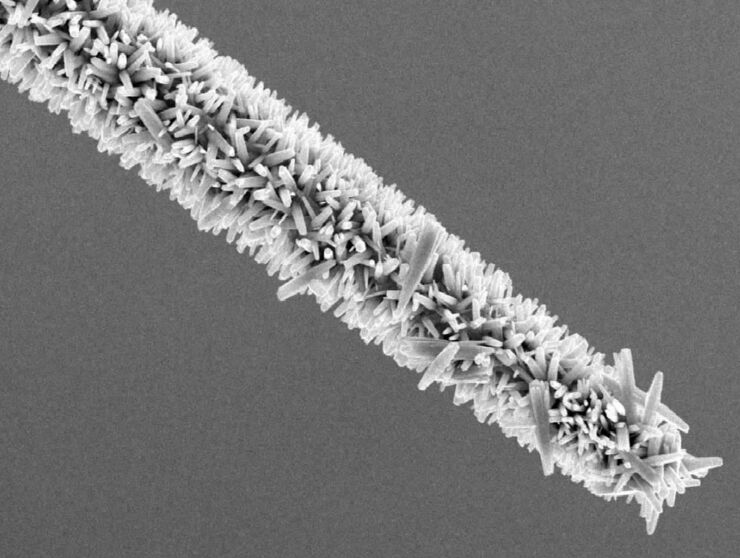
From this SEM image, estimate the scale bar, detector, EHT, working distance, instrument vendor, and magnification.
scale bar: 1000 nm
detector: SEI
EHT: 15 kV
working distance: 7.5 mm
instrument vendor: JEOL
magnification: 3 K X